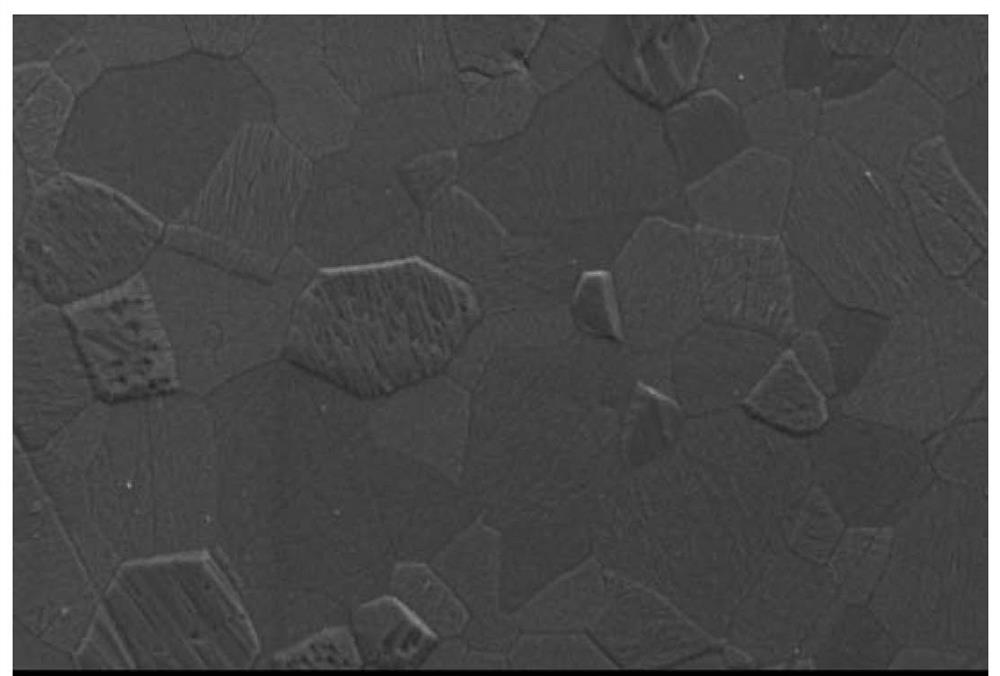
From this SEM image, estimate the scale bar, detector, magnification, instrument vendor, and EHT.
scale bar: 10000 nm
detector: SEI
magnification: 2 K X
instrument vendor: JEOL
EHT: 10 kV